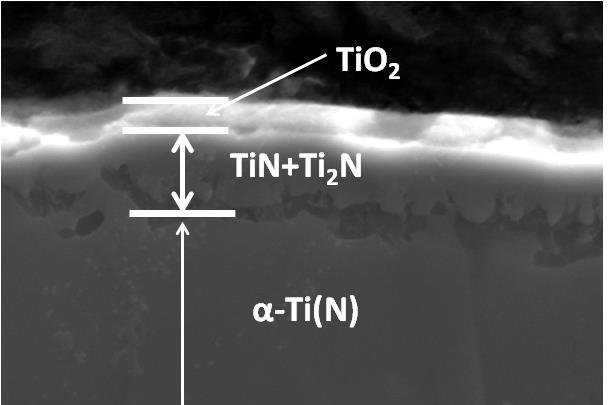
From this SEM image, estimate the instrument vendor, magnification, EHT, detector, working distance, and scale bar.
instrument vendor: Hitachi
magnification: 5 K X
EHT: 20 kV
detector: SE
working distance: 5.5 mm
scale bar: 10000 nm